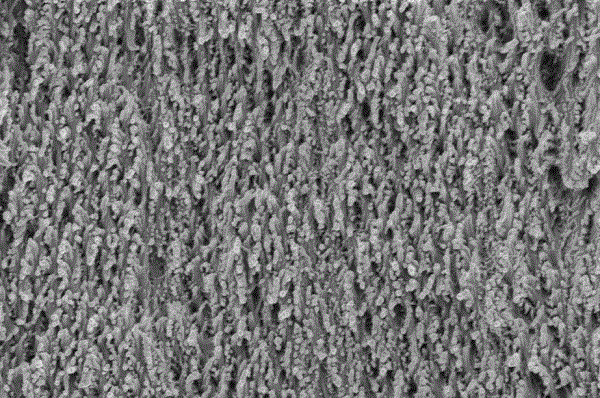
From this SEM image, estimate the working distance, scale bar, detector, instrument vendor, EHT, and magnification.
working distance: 6.3 mm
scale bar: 200 nm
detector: InLens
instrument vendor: Zeiss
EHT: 2 kV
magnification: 20 K X